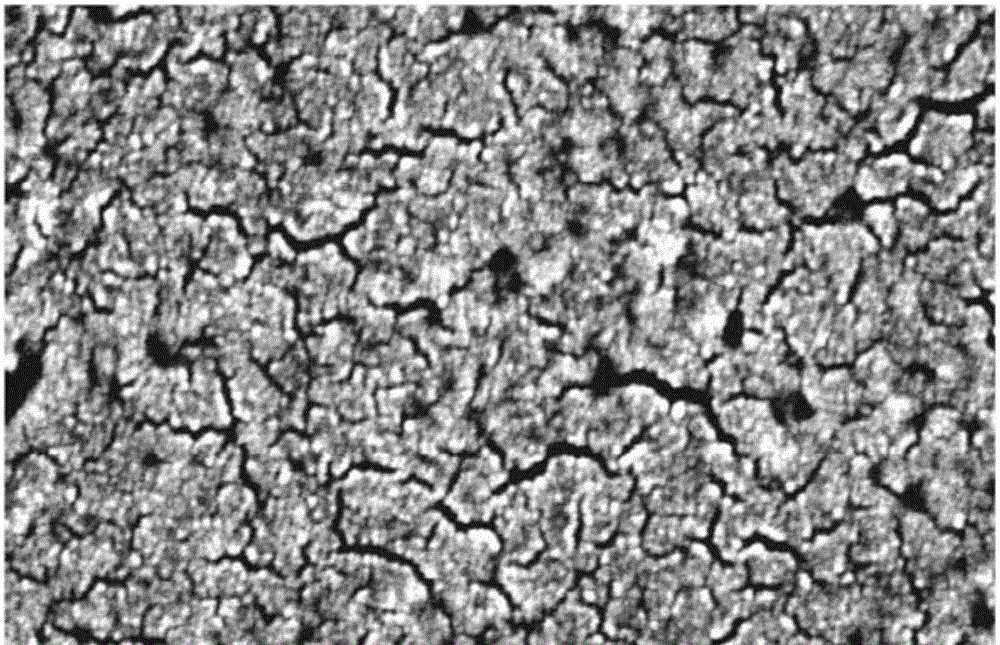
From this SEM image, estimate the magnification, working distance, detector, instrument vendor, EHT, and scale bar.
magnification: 200 K X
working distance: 8.6 mm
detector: SE(M)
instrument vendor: Hitachi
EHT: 5 kV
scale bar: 200 nm